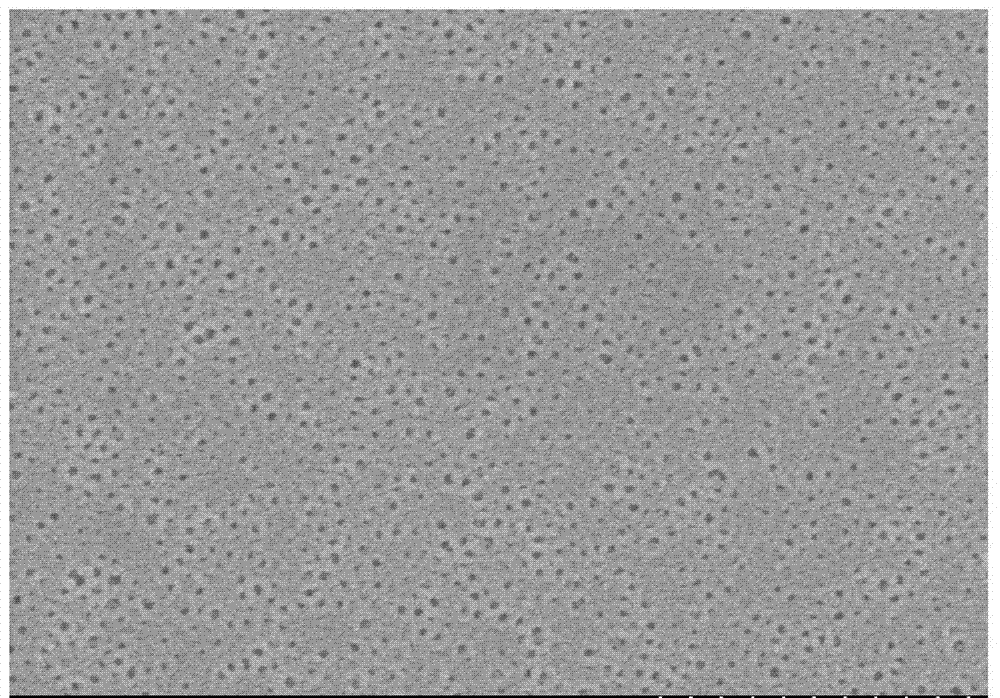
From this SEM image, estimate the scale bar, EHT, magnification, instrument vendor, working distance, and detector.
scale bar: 1000 nm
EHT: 3 kV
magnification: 40 K X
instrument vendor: Hitachi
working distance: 11 mm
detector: SE(M)